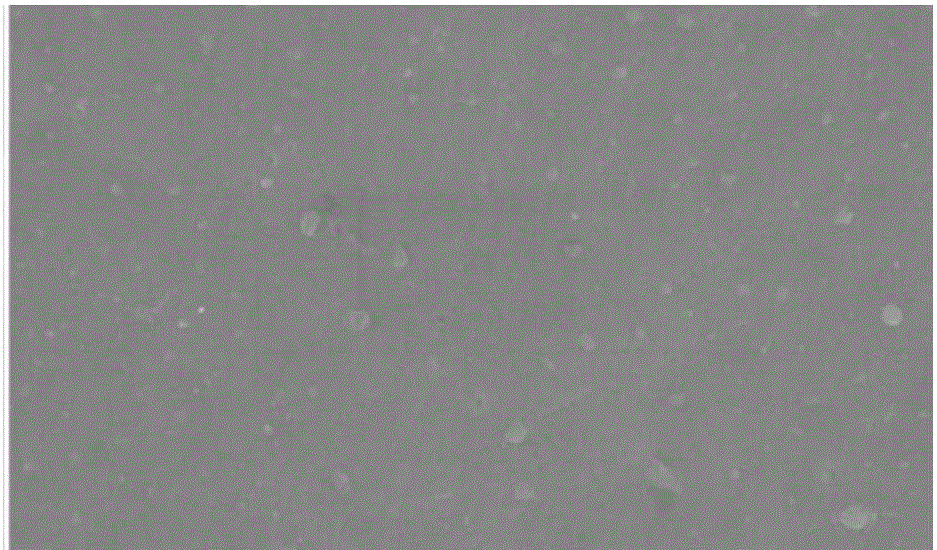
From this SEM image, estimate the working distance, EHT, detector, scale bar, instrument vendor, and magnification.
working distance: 7.7 mm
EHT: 5 kV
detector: SE(M)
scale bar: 5000 nm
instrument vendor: Hitachi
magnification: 10 K X